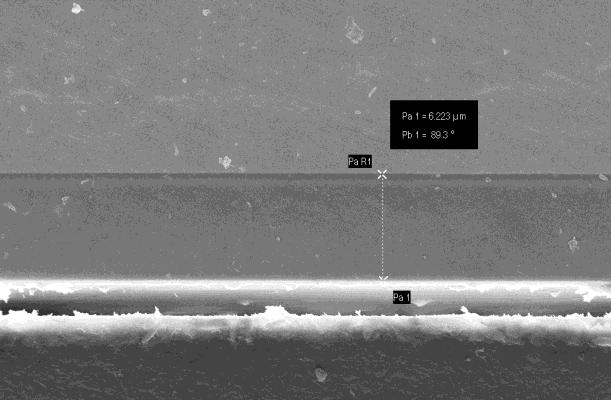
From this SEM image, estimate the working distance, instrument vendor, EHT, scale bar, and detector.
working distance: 9.1 mm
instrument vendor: Zeiss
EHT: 10 kV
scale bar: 2000 nm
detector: InLens